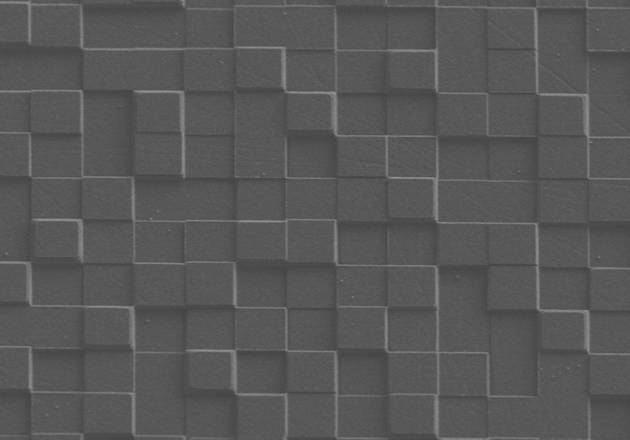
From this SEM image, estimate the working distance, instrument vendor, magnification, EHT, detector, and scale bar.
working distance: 7.8 mm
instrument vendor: Hitachi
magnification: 2 K X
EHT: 3 kV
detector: SE(L)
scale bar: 20000 nm